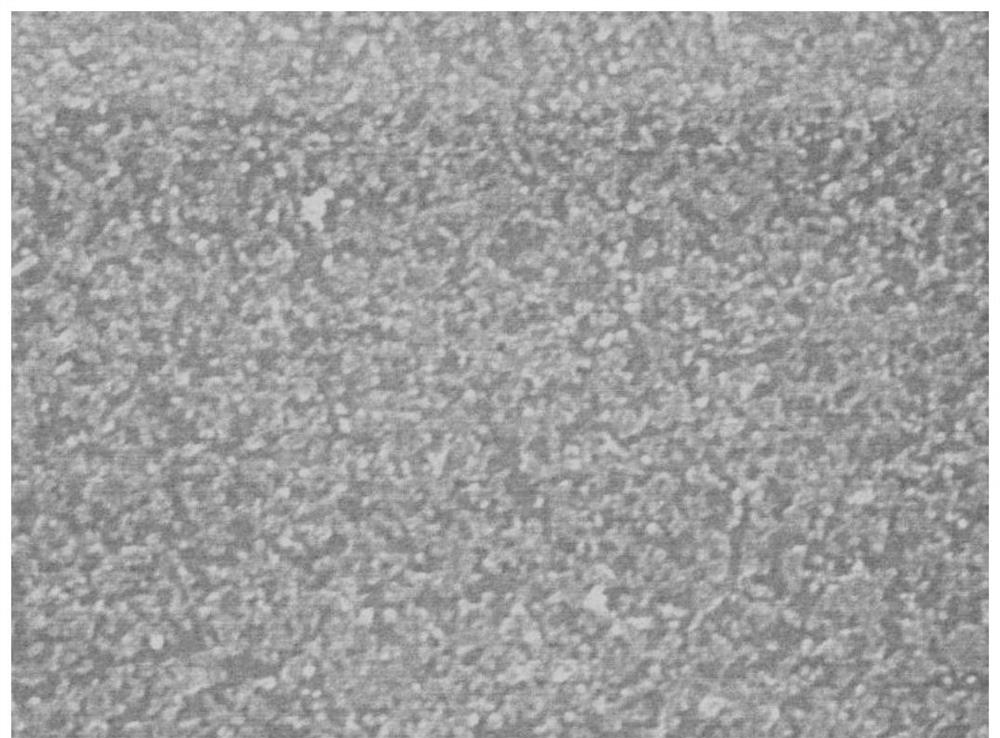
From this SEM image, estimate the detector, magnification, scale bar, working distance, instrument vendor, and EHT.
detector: SEI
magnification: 100 K X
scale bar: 100 nm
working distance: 7.4 mm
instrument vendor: JEOL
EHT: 10 kV